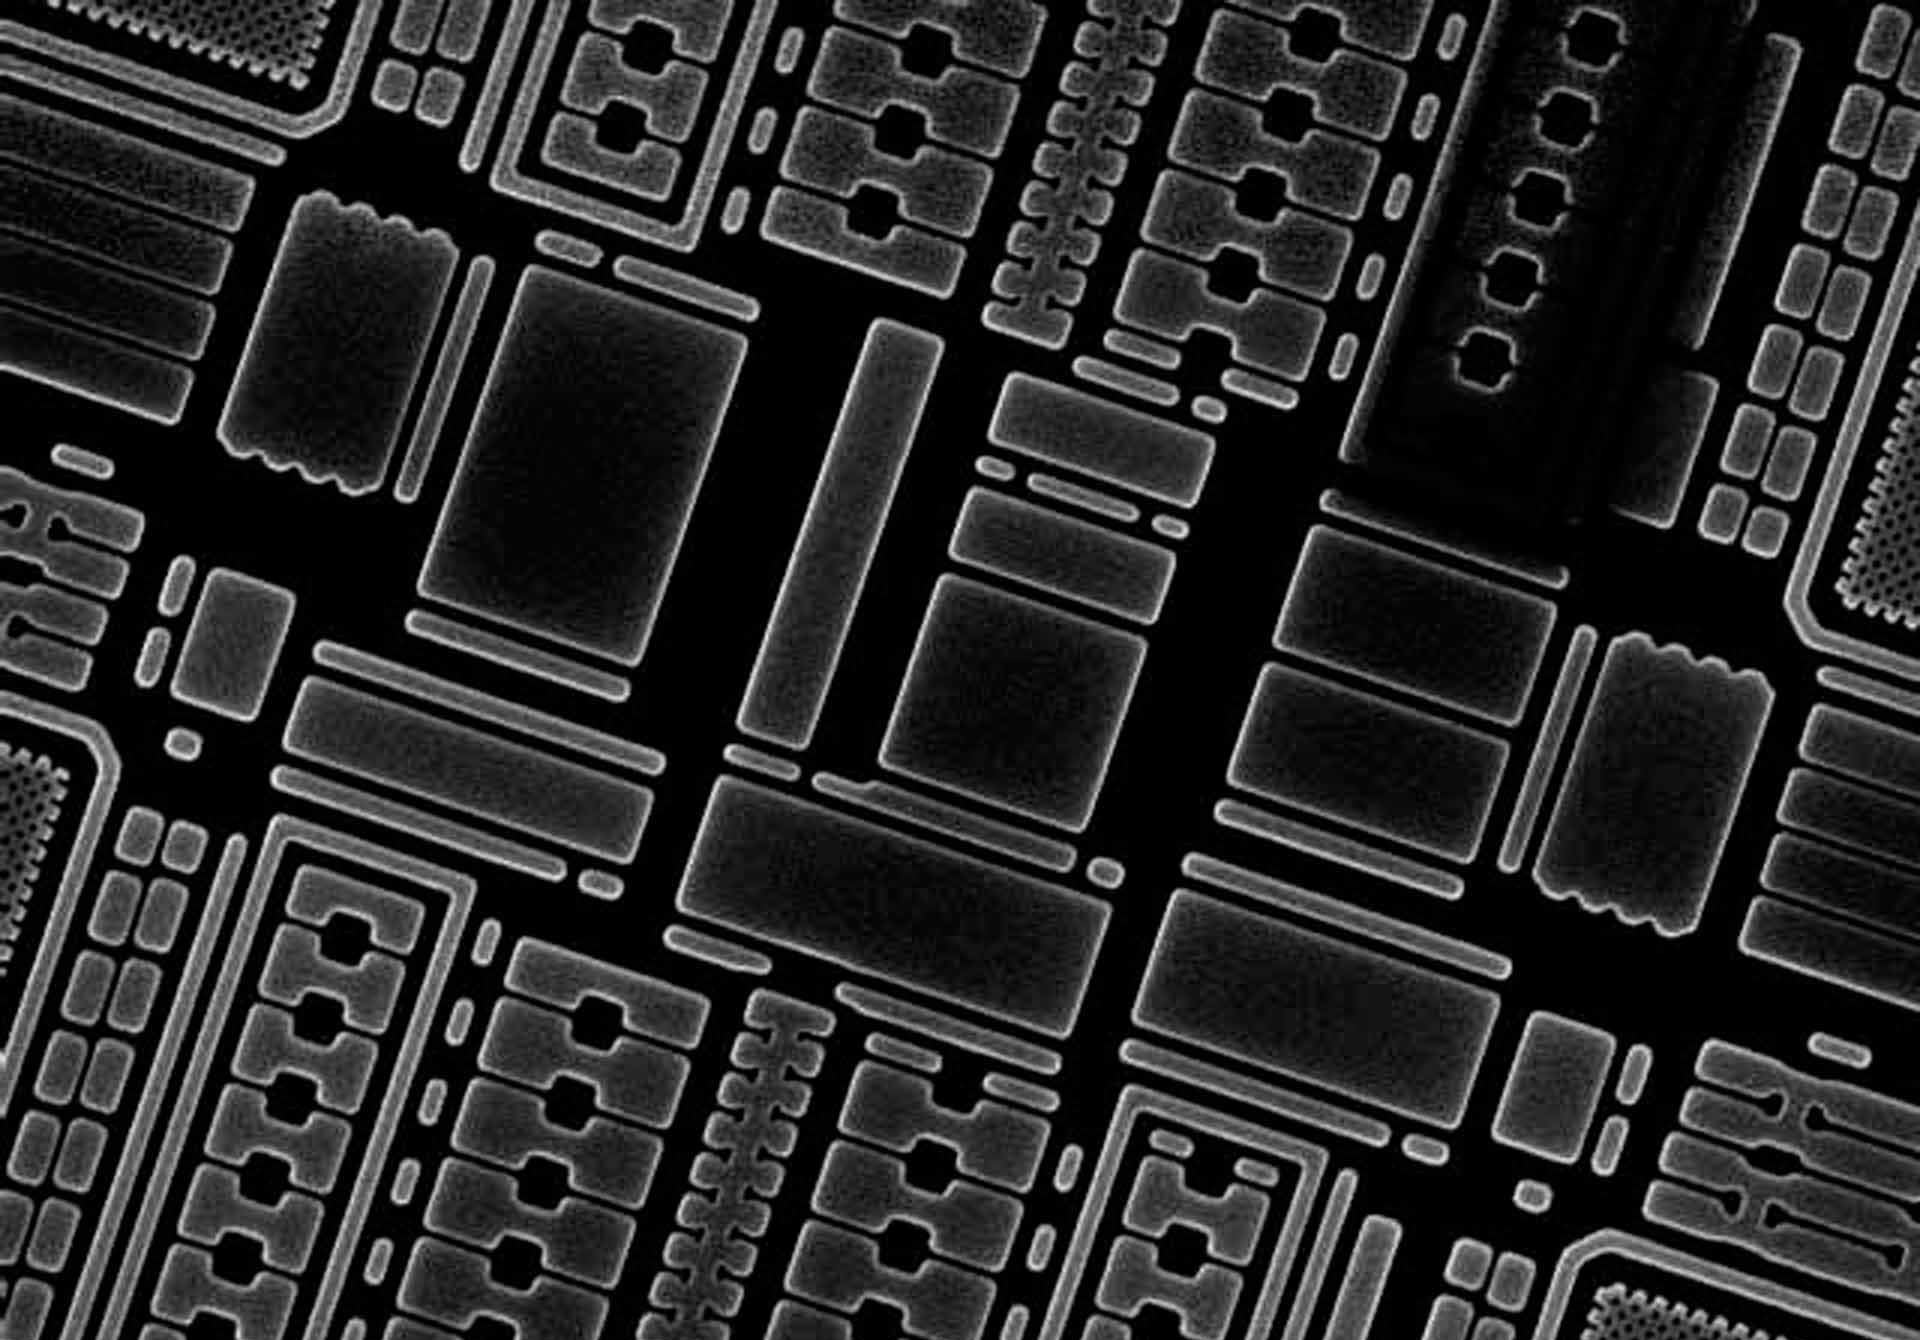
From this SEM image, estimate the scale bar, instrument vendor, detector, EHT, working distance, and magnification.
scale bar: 5000 nm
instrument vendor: Hitachi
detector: SE(M)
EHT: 15 kV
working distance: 18.5 mm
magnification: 9.03 K X